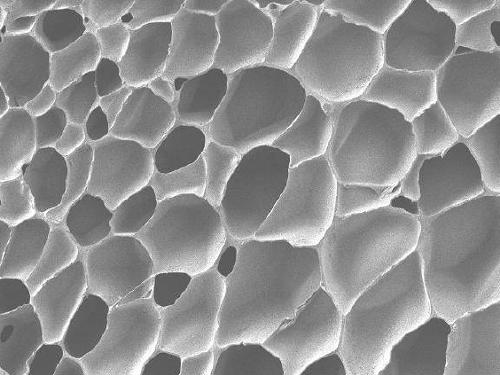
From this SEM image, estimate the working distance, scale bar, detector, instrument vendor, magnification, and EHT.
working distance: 7.2 mm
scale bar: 100000 nm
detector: LEI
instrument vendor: JEOL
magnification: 0.2 K X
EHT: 1 kV